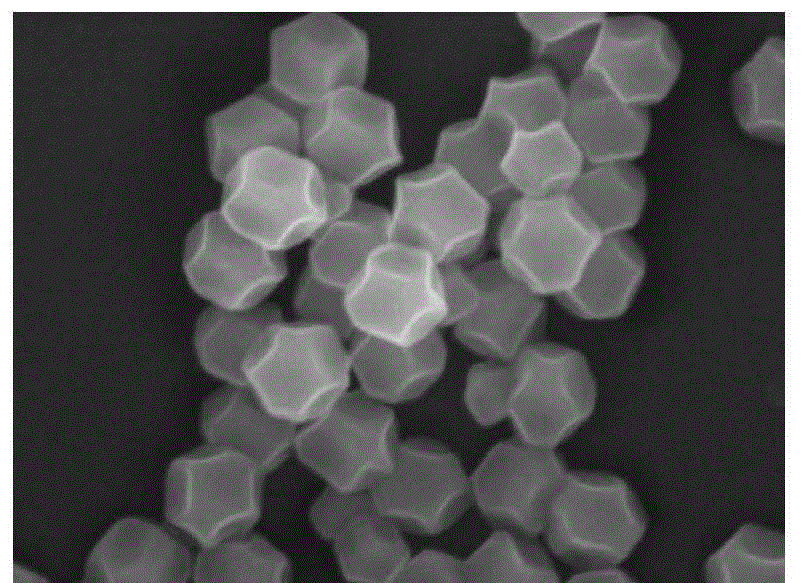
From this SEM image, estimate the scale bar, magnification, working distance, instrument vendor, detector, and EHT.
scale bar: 100 nm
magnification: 75 K X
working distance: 4.2 mm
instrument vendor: JEOL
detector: LED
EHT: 10 kV